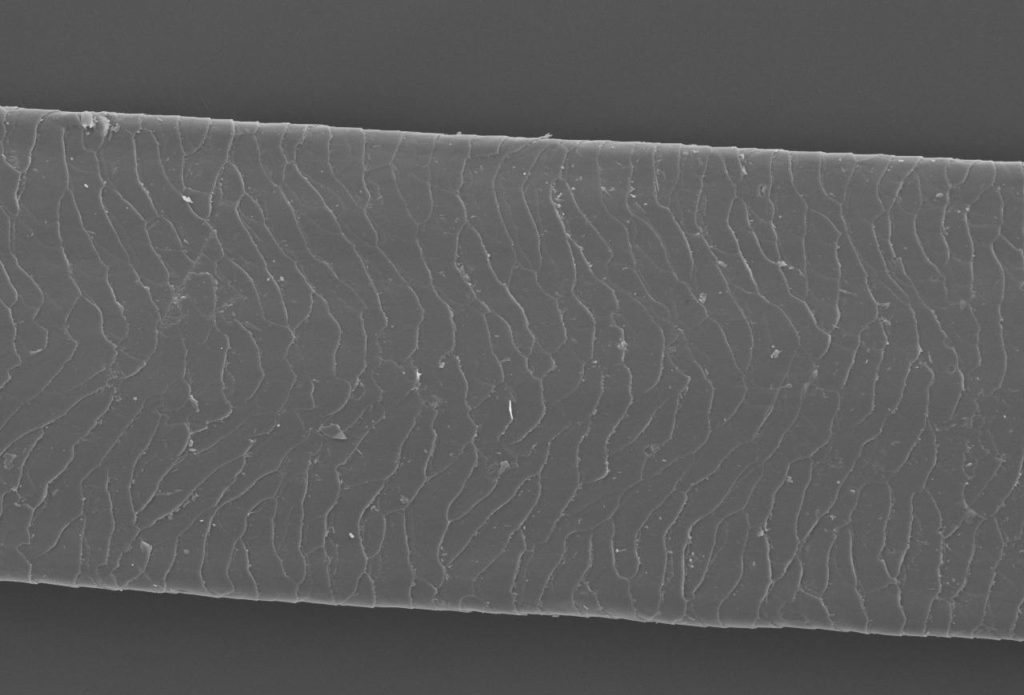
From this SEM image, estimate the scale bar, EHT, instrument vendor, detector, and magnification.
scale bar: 100000 nm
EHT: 10 kV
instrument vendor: JEOL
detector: SEI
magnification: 0.3 K X